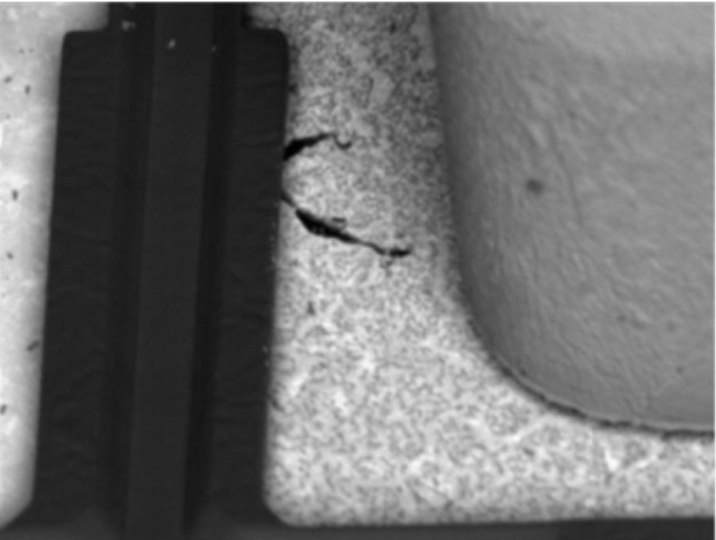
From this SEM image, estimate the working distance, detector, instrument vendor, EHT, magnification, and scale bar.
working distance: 16.3 mm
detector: BSE-COMP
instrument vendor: Hitachi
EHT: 3 kV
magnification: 4 K X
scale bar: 10000 nm